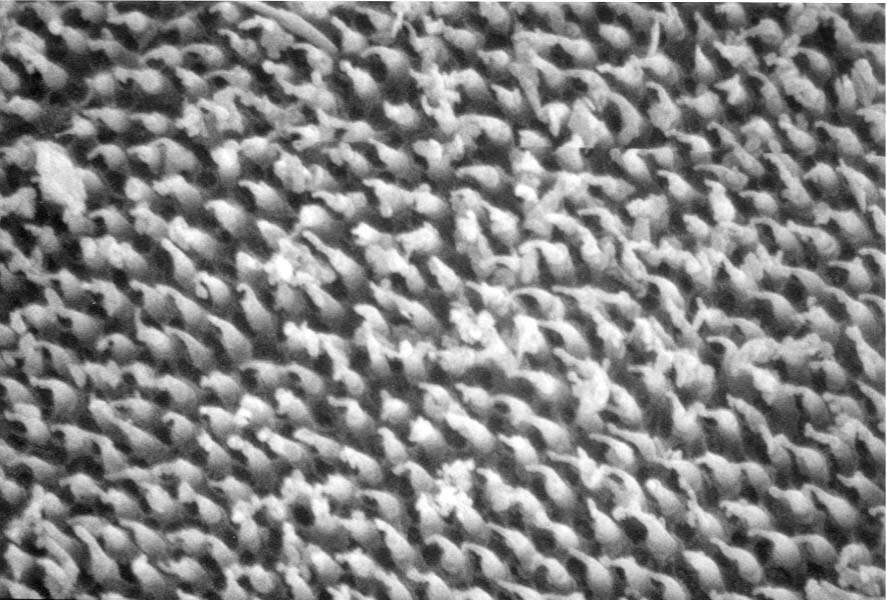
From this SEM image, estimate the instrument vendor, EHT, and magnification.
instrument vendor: JEOL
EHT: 20 kV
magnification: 10 K X